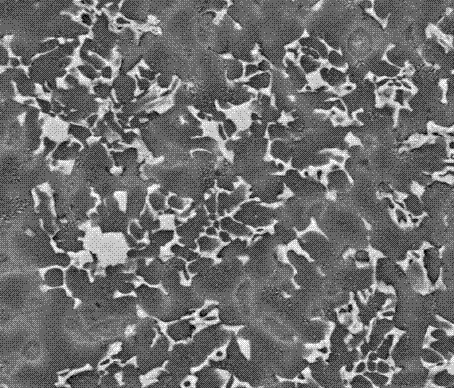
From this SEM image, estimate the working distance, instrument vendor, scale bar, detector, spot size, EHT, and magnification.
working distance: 14.9 mm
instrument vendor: FEI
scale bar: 20000 nm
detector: BSED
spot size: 3.5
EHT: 20 kV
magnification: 2 K X